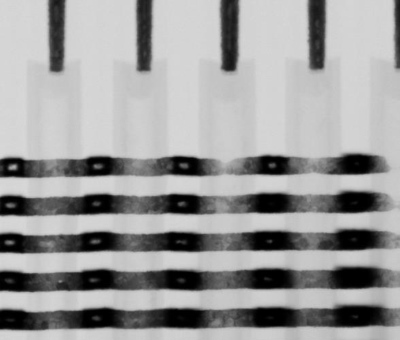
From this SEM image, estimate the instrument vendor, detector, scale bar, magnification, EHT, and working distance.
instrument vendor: FEI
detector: STEM II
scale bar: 300 nm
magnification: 350 K X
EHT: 30 kV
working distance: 4.2 mm